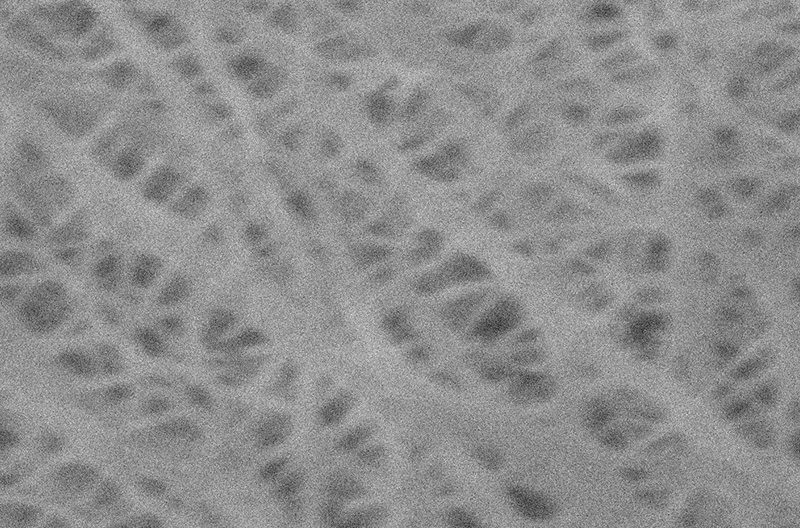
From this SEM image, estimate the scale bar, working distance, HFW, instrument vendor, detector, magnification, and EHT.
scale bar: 500 nm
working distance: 5.77 mm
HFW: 6.41 µm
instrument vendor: other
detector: ETD SE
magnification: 25 K X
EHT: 5 kV